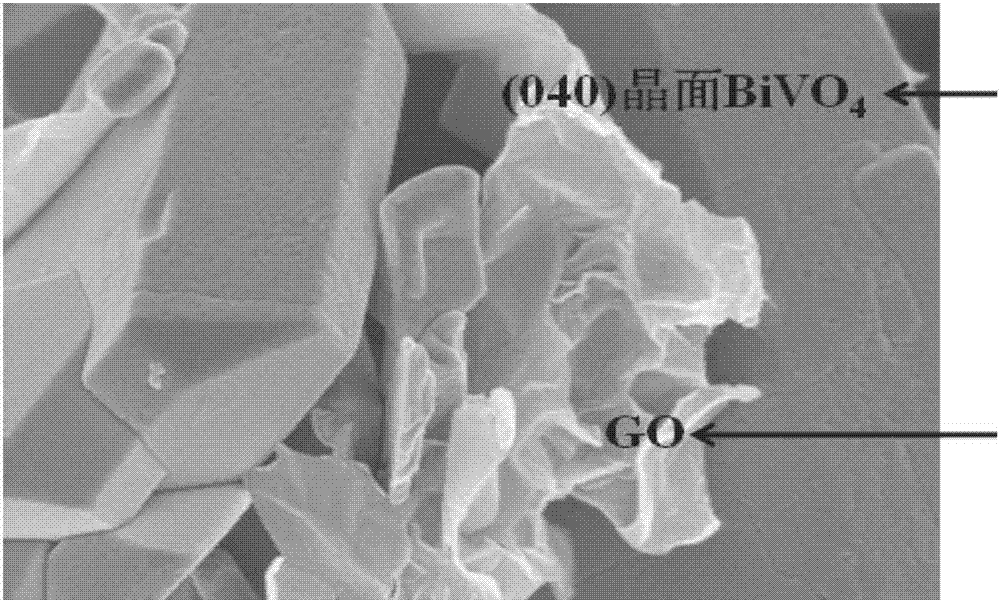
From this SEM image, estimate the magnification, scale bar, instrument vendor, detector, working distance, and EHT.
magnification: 35 K X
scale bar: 1000 nm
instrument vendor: Hitachi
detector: SE(M)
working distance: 9.2 mm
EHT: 3 kV